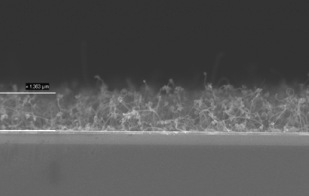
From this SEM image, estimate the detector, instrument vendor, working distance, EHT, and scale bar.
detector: InLens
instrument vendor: Zeiss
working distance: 3 mm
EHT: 5 kV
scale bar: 1000 nm